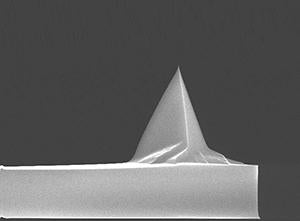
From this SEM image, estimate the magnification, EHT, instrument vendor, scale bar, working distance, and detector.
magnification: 3.5 K X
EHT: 15 kV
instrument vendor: FEI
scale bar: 10000 nm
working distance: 4.6 mm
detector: TLD